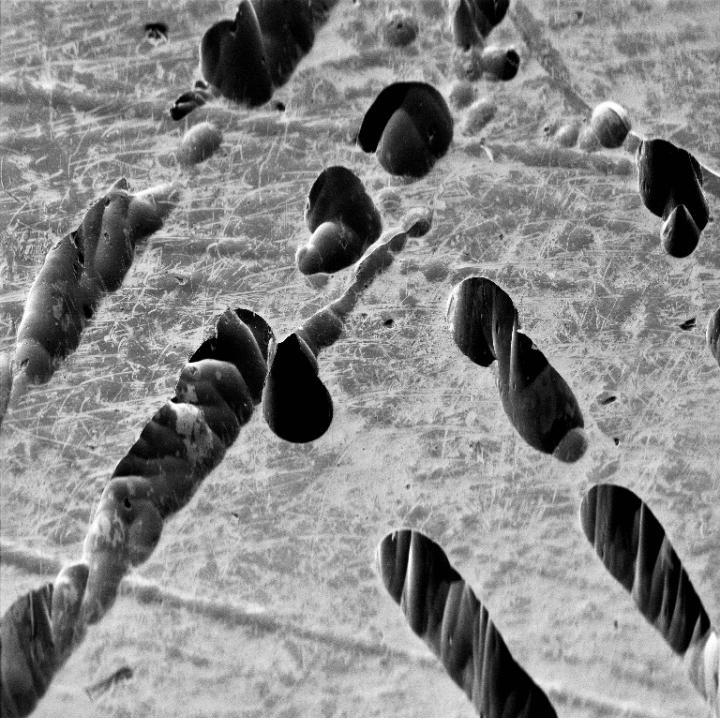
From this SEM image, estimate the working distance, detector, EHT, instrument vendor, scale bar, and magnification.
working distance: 8.98 mm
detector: SE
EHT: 20 kV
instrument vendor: Tescan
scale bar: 500000 nm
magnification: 0.105 K X